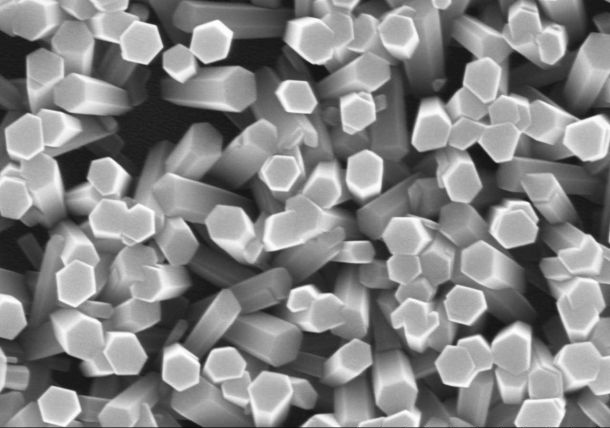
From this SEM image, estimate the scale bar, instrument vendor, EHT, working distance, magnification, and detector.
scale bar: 1000 nm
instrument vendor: Hitachi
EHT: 15 kV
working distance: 8.1 mm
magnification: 50 K X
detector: SE(U)